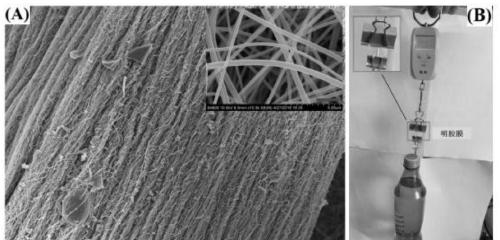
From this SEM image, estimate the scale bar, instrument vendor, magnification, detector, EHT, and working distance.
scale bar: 100000 nm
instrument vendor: Hitachi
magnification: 0.45 K X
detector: SE(M)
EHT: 10 kV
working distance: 8.2 mm